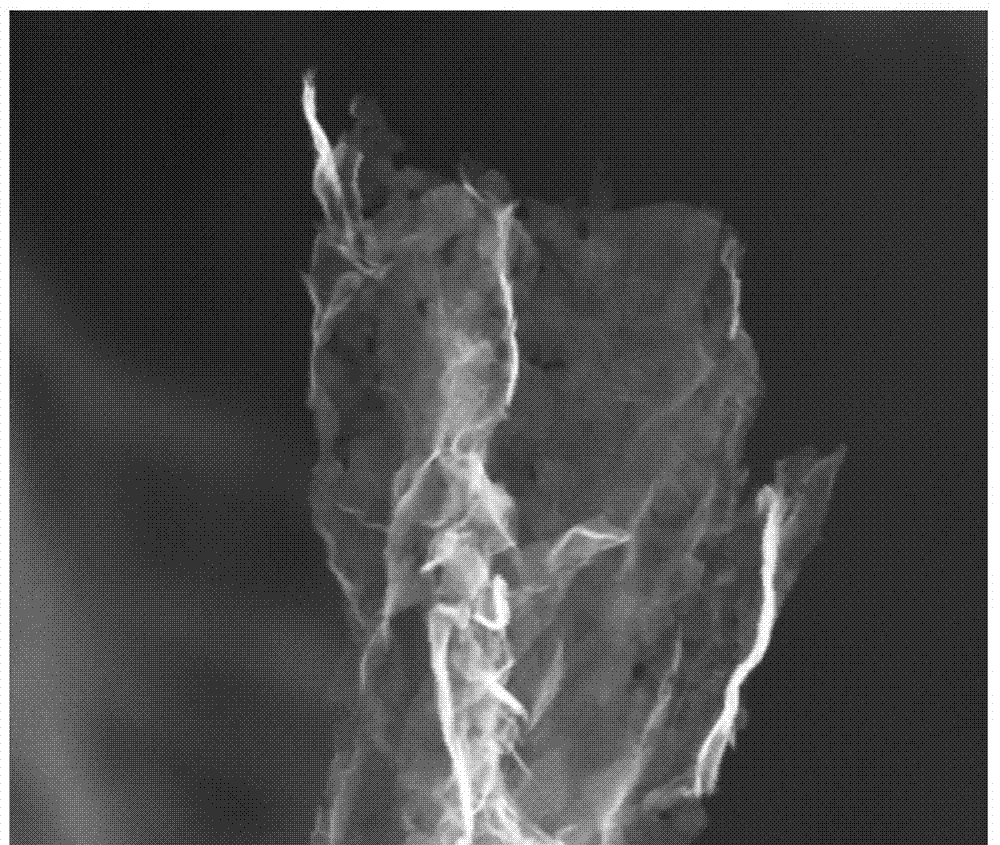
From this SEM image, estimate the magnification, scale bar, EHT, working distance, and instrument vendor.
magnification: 120 K X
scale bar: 500 nm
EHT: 20 kV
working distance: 5.6 mm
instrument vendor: FEI